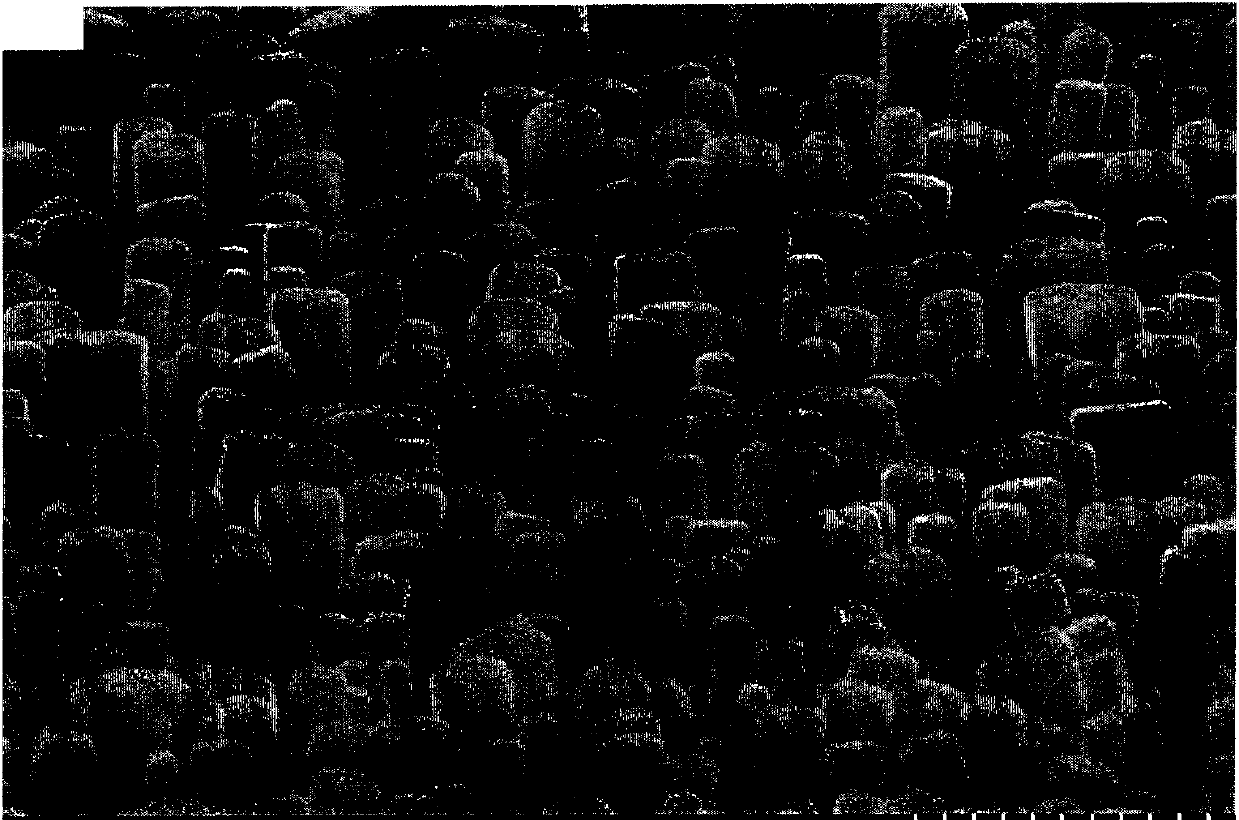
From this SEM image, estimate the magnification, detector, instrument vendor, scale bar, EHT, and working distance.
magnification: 30 K X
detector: SE(M)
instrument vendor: Hitachi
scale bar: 1000 nm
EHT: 15 kV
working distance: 14 mm